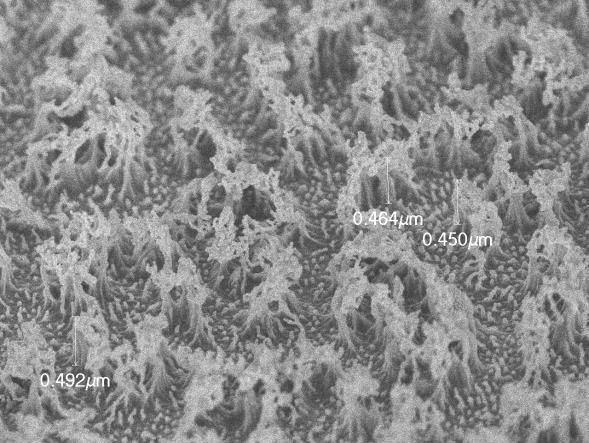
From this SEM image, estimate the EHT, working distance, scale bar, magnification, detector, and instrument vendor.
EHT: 2 kV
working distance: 3.4 mm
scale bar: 1000 nm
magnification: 20 K X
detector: SEI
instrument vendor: JEOL